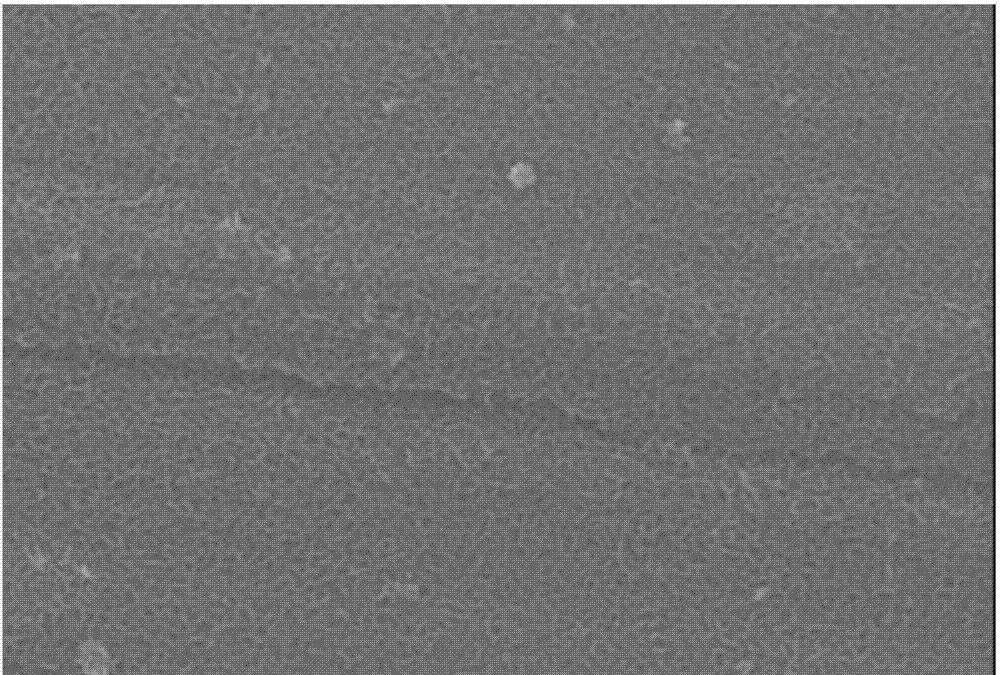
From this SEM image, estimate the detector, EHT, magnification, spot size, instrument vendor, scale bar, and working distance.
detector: SEI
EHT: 20 kV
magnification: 20 K X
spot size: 30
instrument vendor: JEOL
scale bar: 1000 nm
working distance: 11 mm